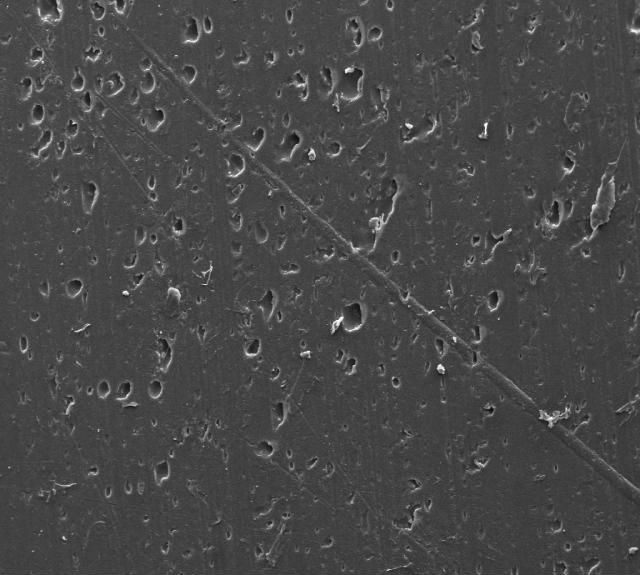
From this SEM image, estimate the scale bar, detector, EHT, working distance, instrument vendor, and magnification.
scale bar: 100000 nm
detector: SE1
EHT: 20 kV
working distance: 15.5 mm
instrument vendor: Zeiss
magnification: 0.2 K X